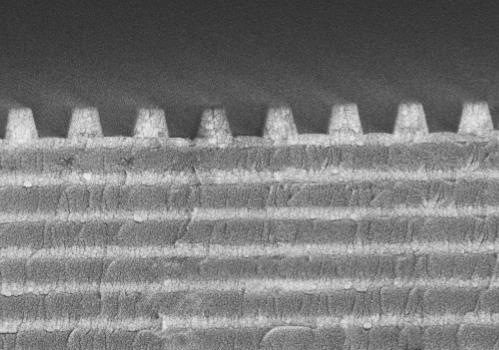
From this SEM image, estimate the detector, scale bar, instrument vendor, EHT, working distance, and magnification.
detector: SEI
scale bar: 1000 nm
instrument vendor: JEOL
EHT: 10 kV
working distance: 6.6 mm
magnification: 20 K X